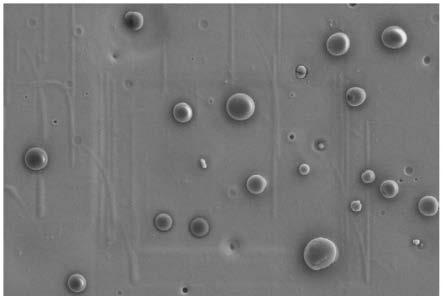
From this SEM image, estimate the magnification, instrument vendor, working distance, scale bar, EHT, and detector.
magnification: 5 K X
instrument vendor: Zeiss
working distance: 7.1 mm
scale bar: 2000 nm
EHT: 3 kV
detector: SE2